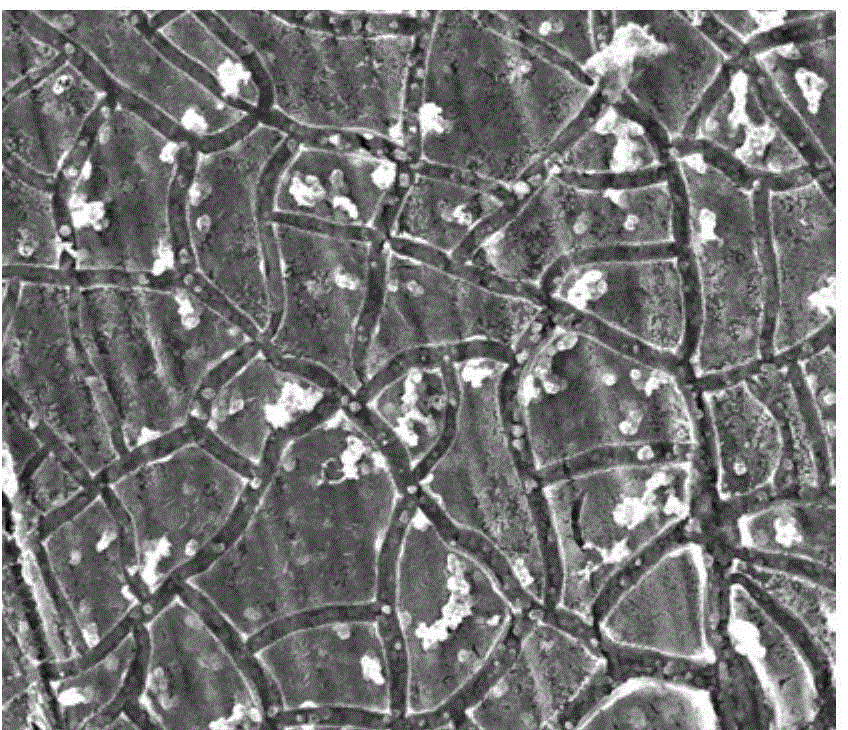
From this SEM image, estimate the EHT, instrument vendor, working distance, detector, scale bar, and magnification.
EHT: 25 kV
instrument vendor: FEI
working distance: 10.7 mm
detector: SE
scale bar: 20000 nm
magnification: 5 K X